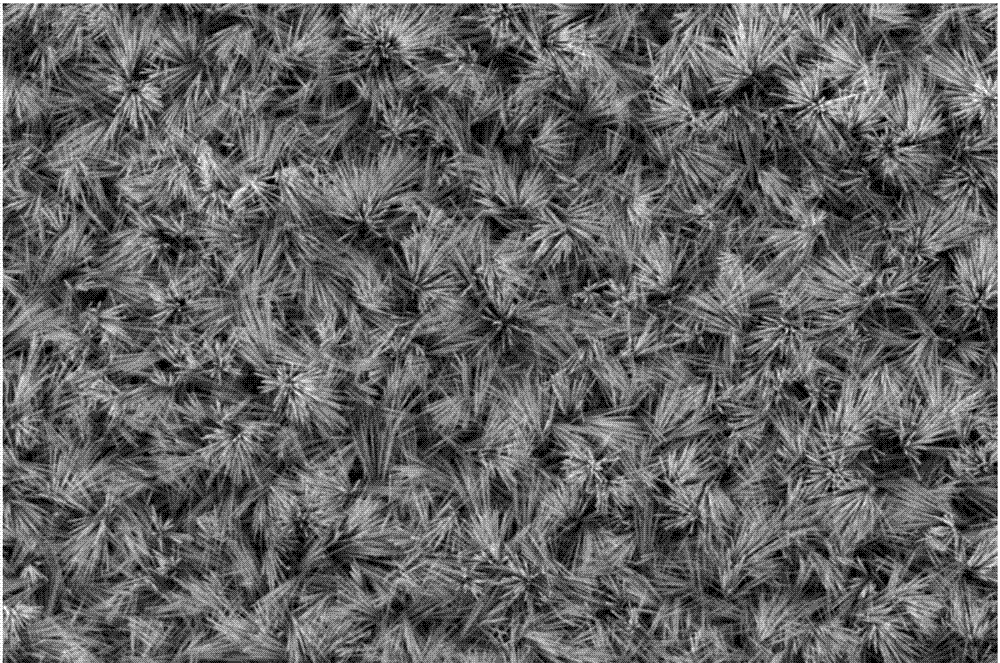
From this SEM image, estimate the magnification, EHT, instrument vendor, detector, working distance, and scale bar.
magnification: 2.59 K X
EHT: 10 kV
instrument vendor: FEI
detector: ETD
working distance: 9.3 mm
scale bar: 40000 nm